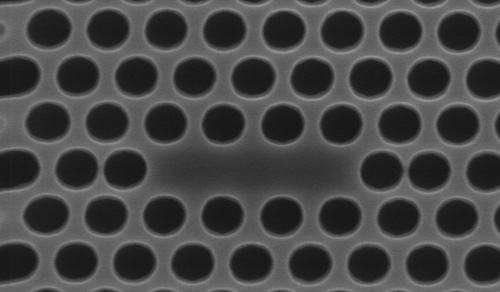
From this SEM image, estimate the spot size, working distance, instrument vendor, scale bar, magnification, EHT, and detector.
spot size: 3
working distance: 4.7 mm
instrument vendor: FEI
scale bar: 500 nm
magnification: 56.821 K X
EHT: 5 kV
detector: TLD